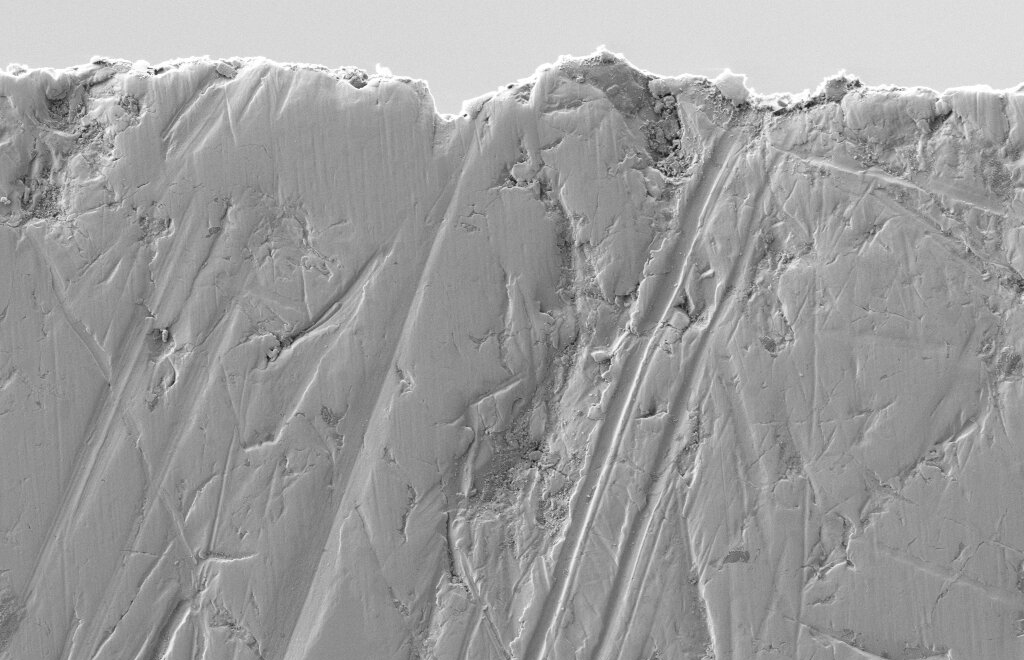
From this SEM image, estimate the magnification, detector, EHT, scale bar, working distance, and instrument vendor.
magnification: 5 K X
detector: SE2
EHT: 1 kV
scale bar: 1000 nm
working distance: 5.2 mm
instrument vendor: Zeiss